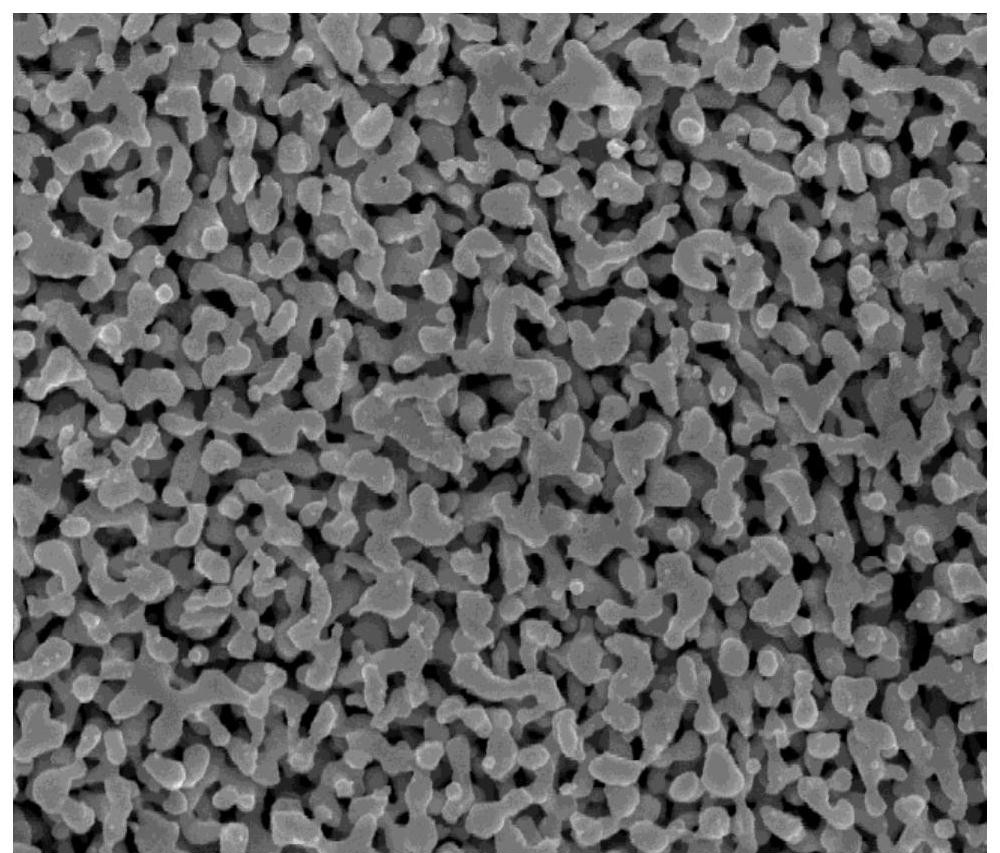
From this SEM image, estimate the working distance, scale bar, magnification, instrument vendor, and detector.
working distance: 6.6 mm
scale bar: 2000 nm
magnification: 40 K X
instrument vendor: FEI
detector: SE